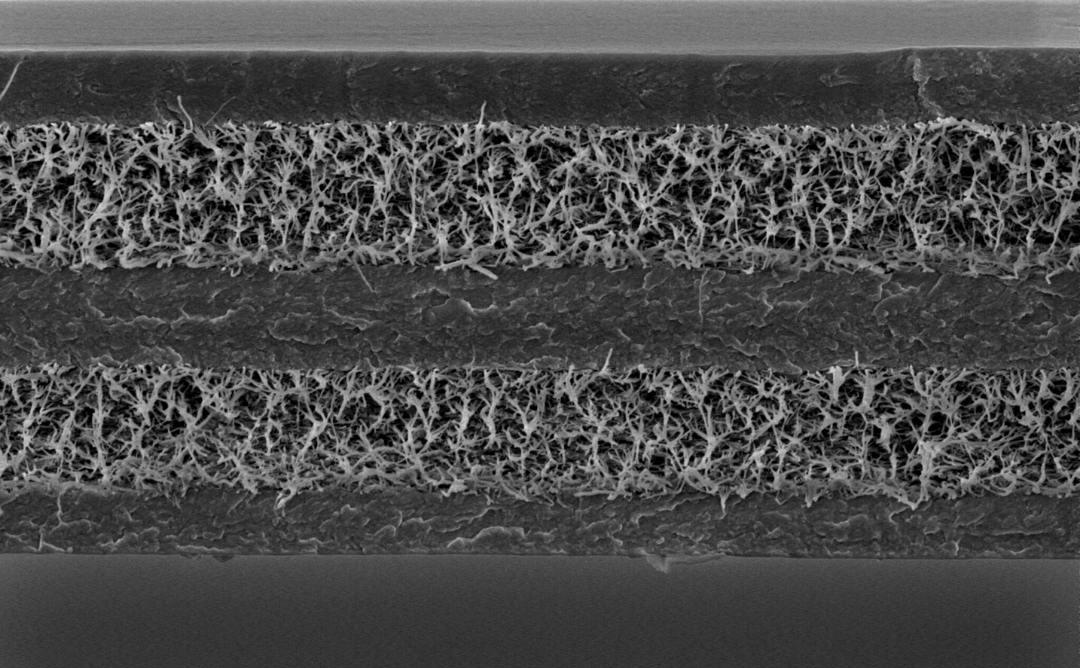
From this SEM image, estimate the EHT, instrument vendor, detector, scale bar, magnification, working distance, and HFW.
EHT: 5 kV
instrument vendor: other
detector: SED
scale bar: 15000 nm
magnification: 10 K X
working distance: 8.471 mm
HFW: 51.9 µm